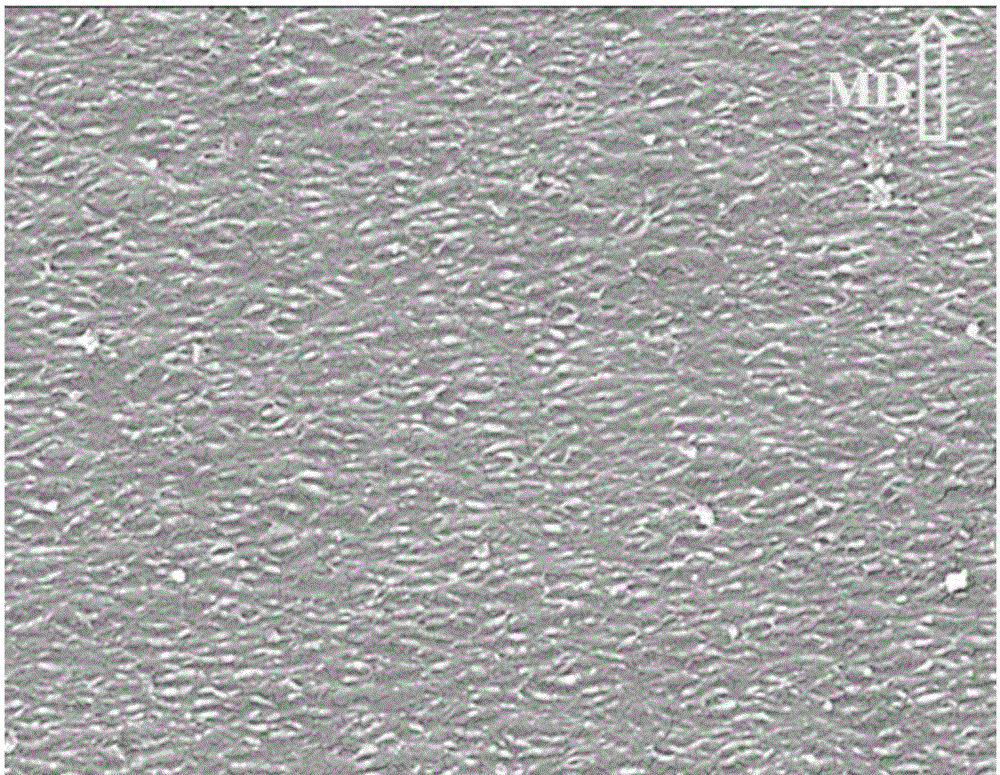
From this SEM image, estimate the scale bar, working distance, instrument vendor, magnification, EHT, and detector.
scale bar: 5000 nm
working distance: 8.8 mm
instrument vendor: Hitachi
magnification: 10 K X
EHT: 5 kV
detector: SE(M,LA0)